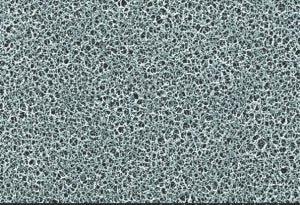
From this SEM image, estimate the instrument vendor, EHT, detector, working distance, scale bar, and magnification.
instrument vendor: JEOL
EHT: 15 kV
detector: SE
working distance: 32.7 mm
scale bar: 30000 nm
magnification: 1 K X